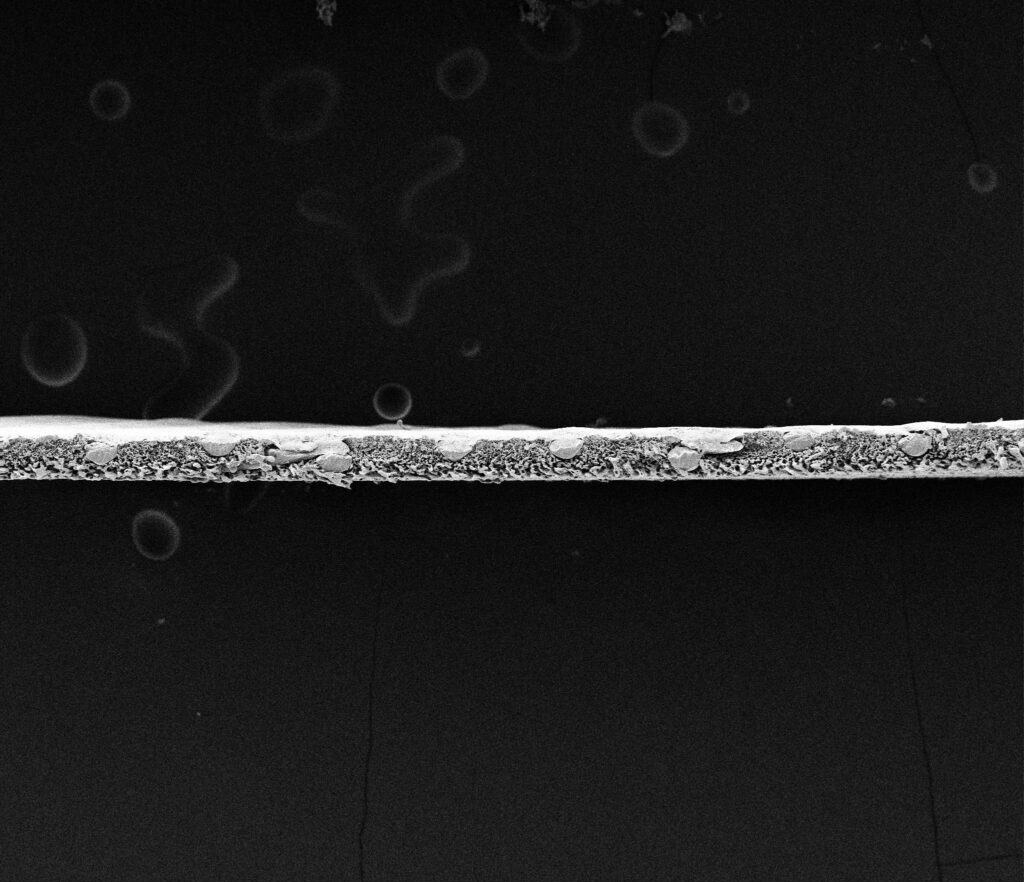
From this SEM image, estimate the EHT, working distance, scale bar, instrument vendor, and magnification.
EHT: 5 kV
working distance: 5.3 mm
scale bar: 400000 nm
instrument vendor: FEI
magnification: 0.1 K X